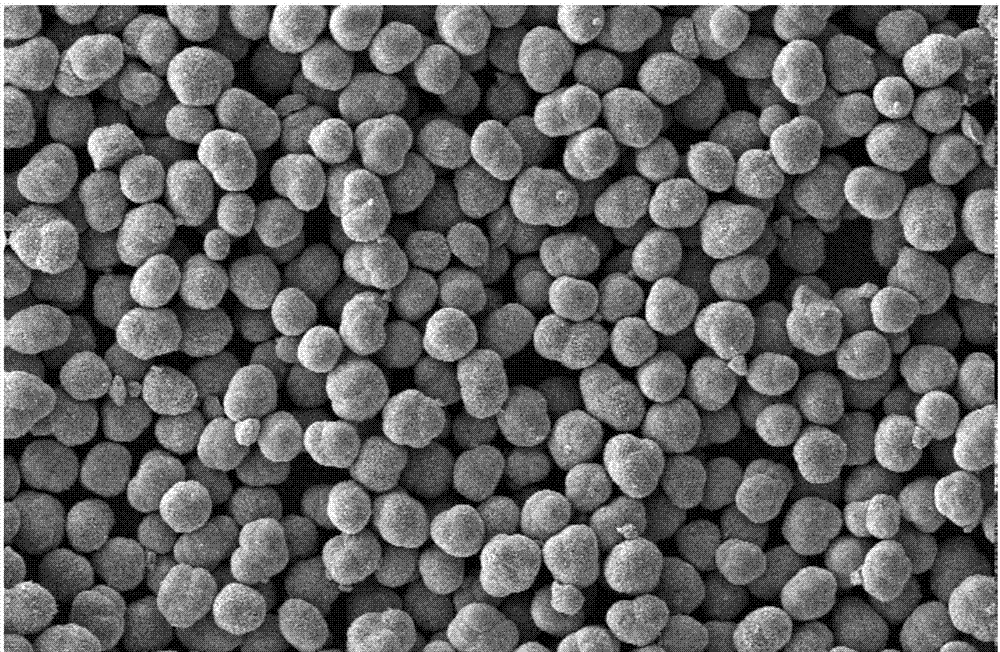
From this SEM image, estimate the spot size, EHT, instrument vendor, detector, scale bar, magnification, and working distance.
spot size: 3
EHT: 10 kV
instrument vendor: FEI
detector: SE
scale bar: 50000 nm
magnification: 0.4 K X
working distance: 9.8 mm